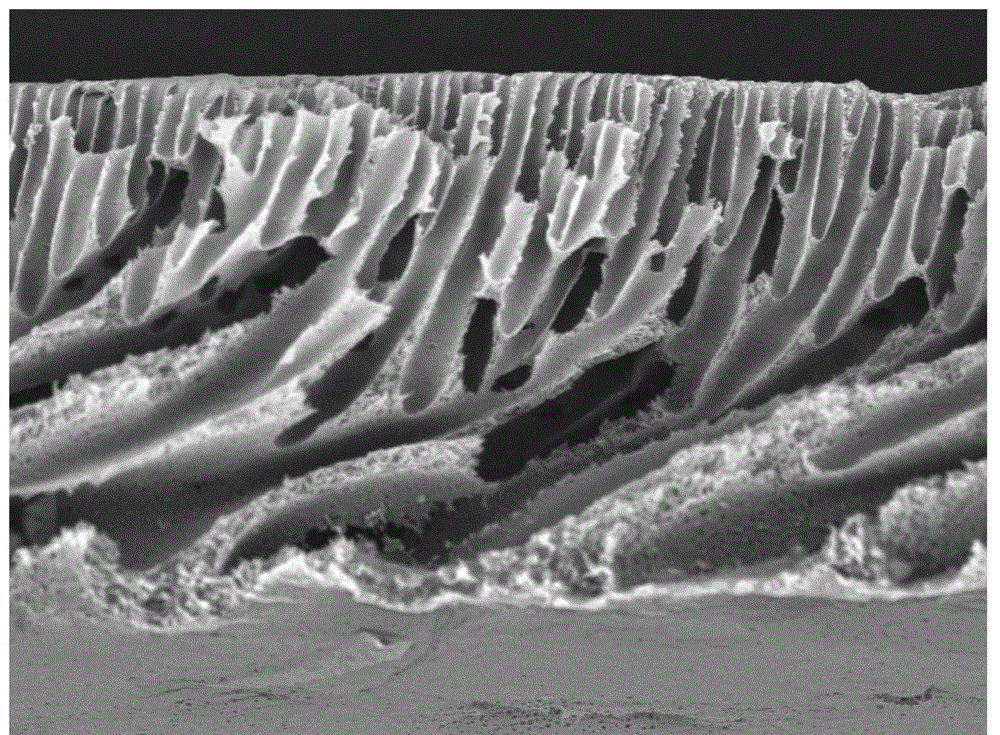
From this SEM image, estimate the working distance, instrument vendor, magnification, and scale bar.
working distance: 4.8 mm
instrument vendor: Hitachi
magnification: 1 K X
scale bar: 100000 nm